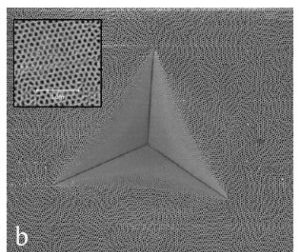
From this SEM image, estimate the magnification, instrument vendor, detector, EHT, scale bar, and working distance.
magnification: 17.3 K X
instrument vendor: FEI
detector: TLD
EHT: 2 kV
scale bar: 10000 nm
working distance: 4.3 mm